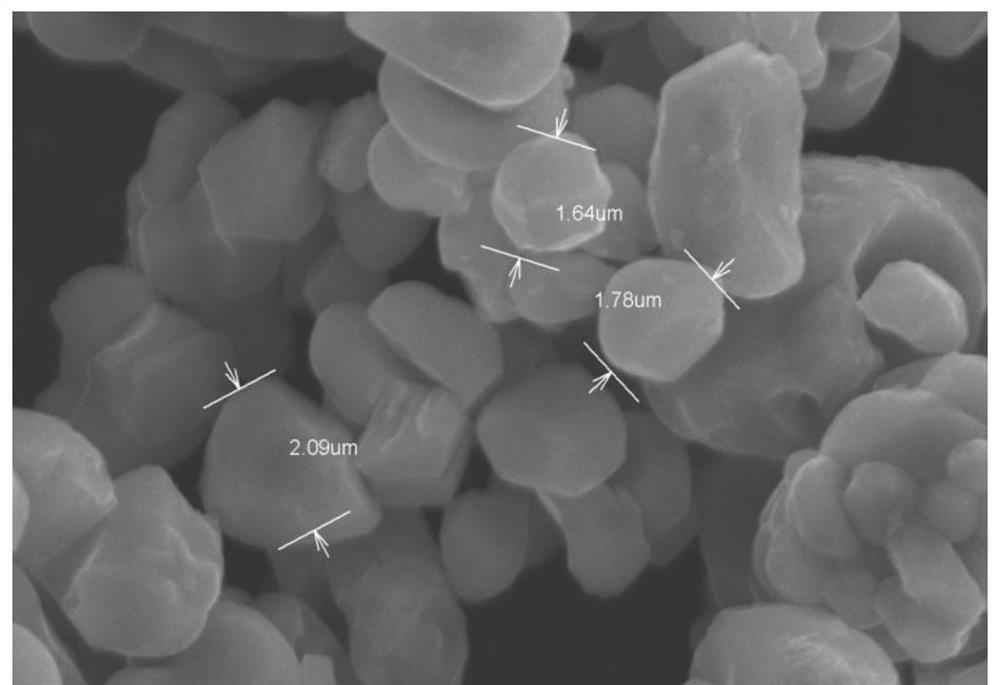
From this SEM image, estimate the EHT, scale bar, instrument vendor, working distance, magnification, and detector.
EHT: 15 kV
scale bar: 5000 nm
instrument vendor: Hitachi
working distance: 10.3 mm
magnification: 10 K X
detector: SE